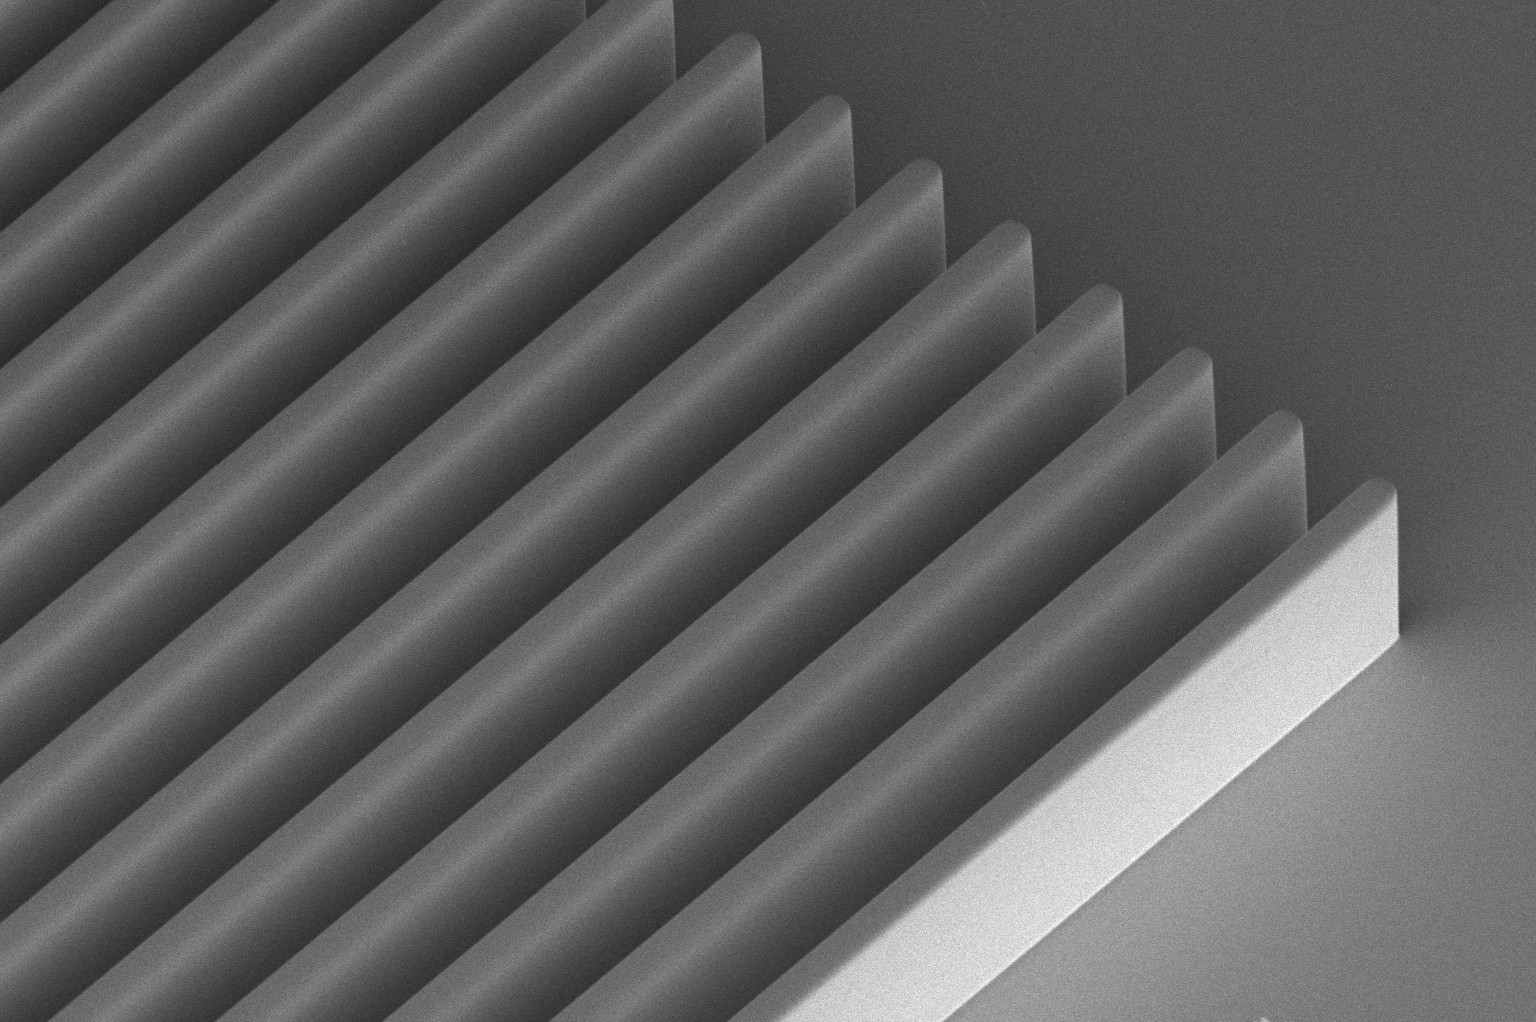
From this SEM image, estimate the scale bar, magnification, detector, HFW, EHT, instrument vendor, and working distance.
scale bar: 50000 nm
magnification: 0.65 K X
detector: TLD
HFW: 319 µm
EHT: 2 kV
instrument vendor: FEI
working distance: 4.1 mm